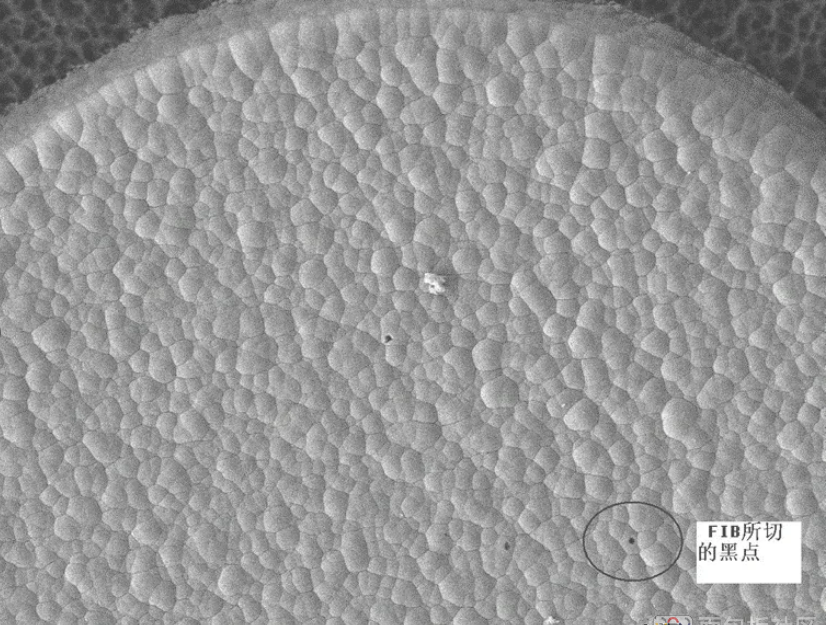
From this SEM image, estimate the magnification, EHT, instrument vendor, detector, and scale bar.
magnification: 0.8 K X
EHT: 10 kV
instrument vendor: JEOL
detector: LEI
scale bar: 10000 nm